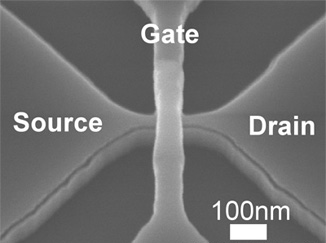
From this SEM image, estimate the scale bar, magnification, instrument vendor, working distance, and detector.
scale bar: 500 nm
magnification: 100 K X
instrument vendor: Hitachi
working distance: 7.7 mm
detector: SE(U)